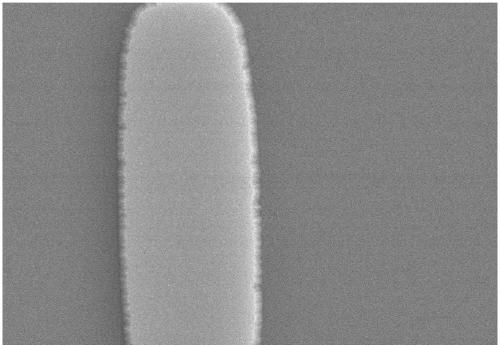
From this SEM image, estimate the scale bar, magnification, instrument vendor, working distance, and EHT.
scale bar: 5000 nm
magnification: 10 K X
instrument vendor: Hitachi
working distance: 11.2 mm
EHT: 15 kV